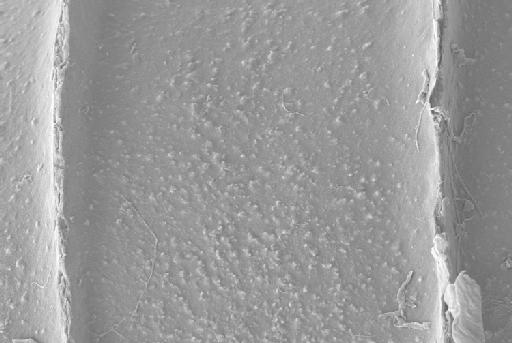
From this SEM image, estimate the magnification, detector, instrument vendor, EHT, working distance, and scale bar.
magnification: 4.09 K X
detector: SE2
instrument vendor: Zeiss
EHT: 5 kV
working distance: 4.4 mm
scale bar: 10000 nm